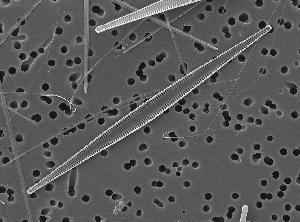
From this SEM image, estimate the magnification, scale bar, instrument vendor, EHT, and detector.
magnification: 1.2 K X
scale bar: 10000 nm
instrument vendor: JEOL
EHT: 10 kV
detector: SEI